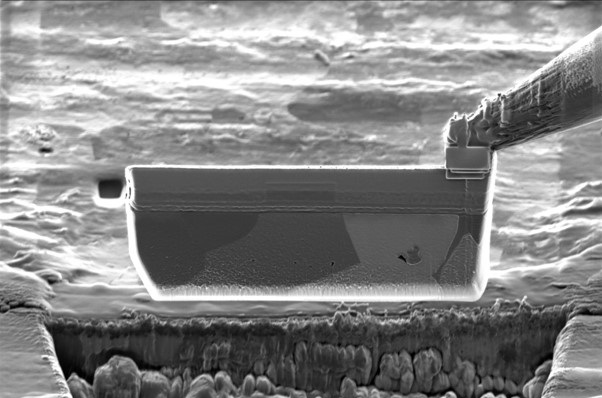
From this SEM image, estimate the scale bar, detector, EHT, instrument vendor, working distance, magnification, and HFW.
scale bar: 3000 nm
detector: InLens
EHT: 1.8 kV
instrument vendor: Zeiss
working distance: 5.2 mm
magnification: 2.88 K X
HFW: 39.74 µm